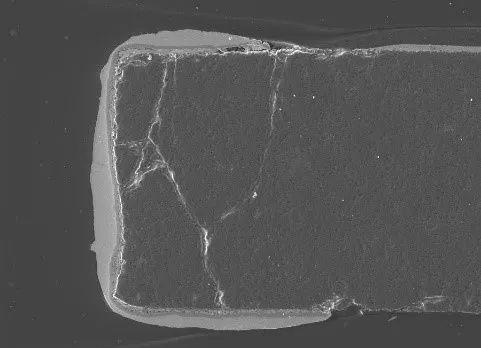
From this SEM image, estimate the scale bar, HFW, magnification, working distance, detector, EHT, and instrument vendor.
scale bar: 200000 nm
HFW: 716 µm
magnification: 0.387 K X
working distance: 20.22 mm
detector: SE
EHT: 20 kV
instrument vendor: Tescan